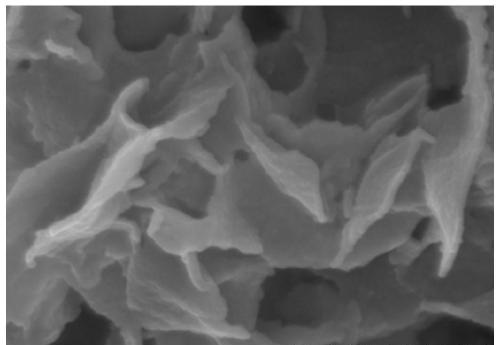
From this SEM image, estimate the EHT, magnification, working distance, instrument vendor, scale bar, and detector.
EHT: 15 kV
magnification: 100 K X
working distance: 6.3 mm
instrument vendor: Hitachi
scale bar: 500 nm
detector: SE(U)